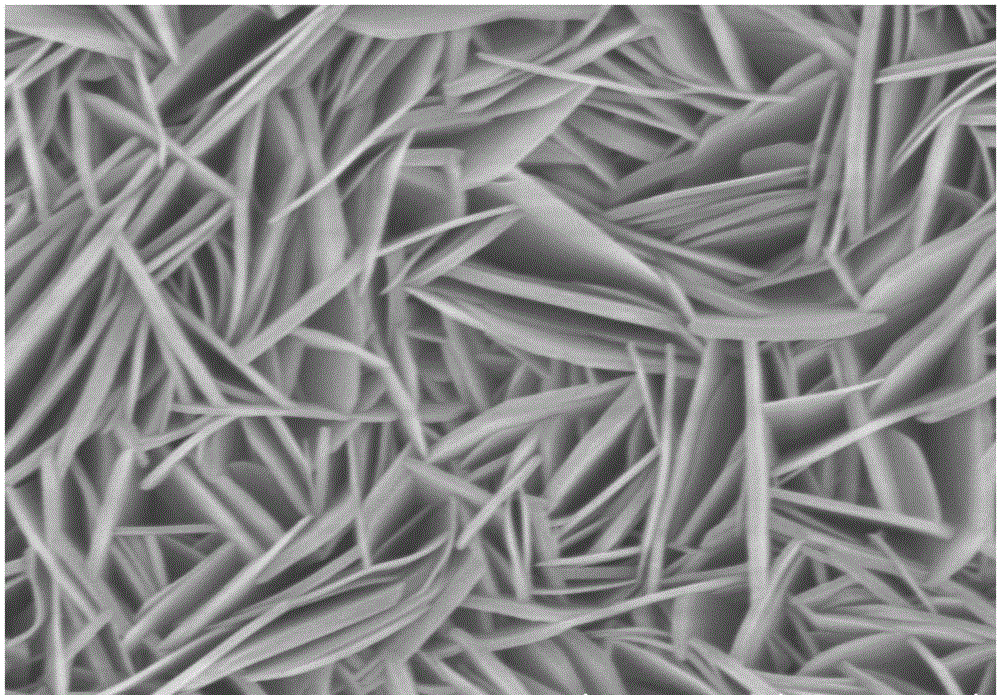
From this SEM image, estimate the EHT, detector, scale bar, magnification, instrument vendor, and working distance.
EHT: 5 kV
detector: SE(UL)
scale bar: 2000 nm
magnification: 25 K X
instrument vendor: Hitachi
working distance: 9.3 mm